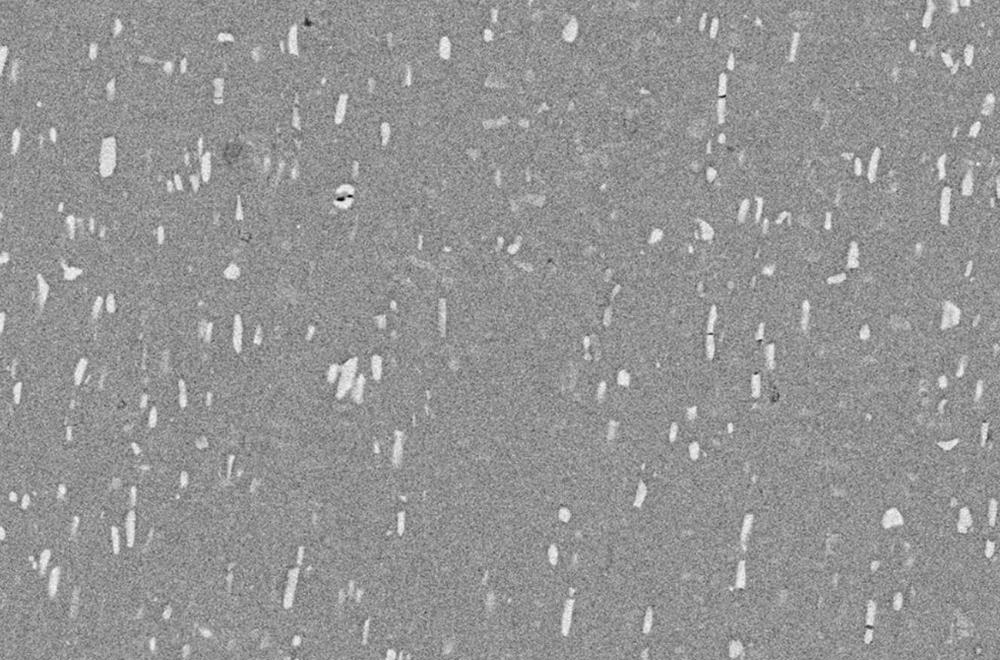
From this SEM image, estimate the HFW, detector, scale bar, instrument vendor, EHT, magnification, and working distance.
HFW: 254 µm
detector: T1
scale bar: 50000 nm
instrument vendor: Thermo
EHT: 20 kV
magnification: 0.5 K X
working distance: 12.8 mm